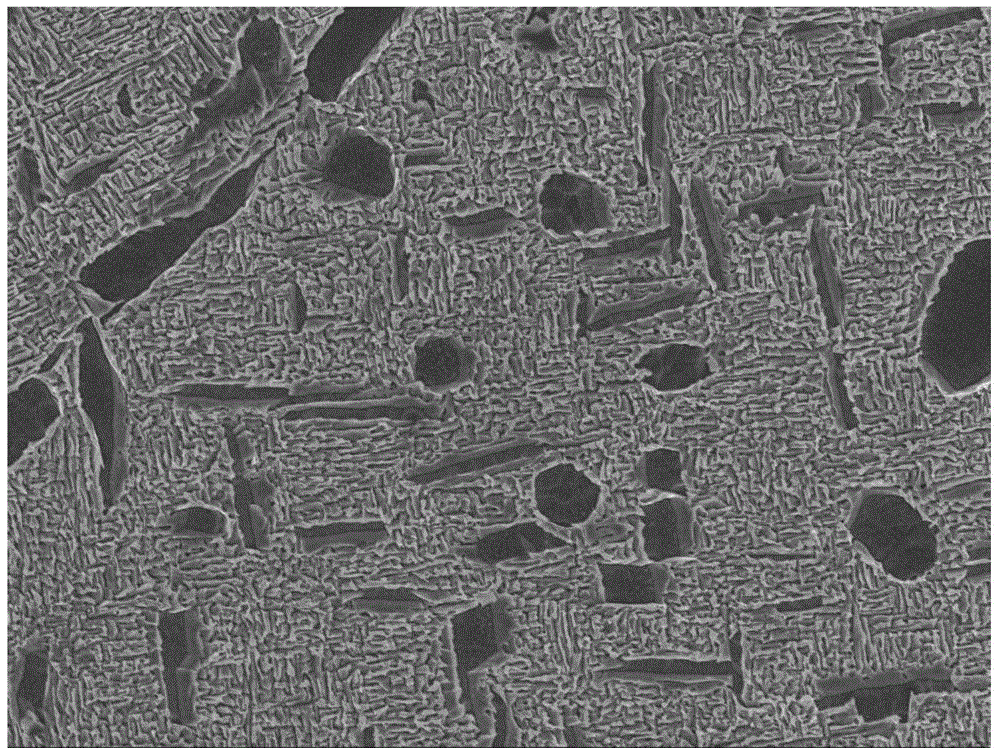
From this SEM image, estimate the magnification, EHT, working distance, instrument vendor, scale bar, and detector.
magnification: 5 K X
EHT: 5 kV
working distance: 9 mm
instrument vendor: JEOL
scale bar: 1000 nm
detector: SEI